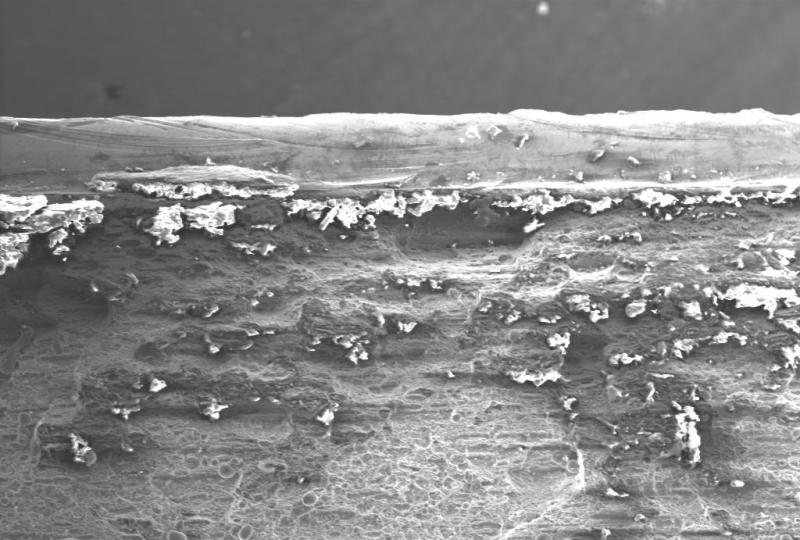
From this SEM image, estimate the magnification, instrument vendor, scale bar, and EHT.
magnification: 0.1 K X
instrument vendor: other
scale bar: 1000000 nm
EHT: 20 kV